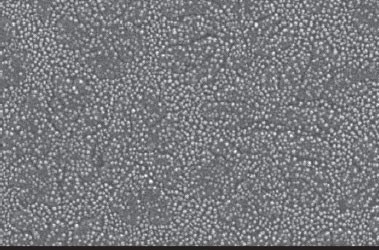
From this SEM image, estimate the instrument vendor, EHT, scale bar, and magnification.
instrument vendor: JEOL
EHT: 20 kV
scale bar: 500 nm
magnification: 40 K X